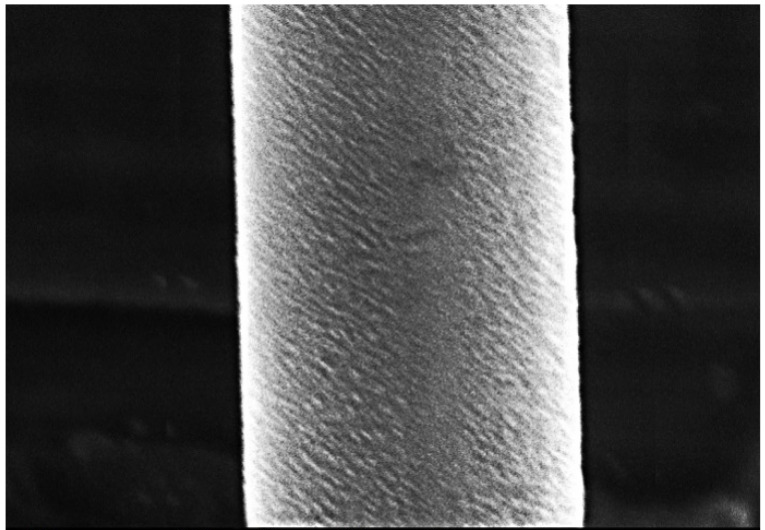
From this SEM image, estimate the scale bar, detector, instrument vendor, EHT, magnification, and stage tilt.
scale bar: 10000 nm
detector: SED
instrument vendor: other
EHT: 20 kV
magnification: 2.5 K X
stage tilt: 0°